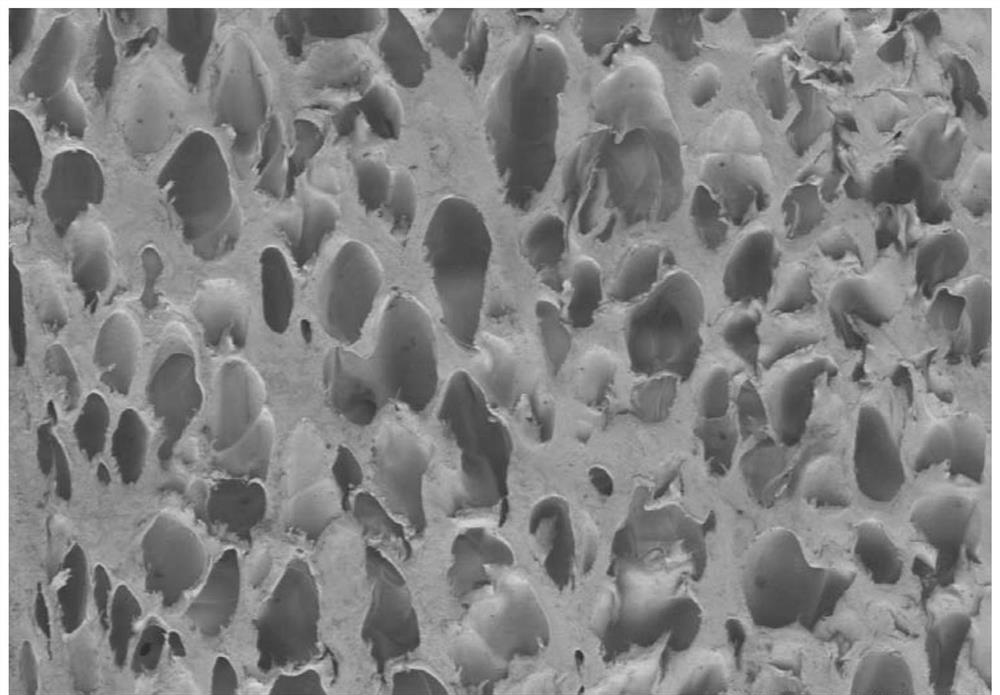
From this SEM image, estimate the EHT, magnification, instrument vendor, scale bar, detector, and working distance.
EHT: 1 kV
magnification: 0.03 K X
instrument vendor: Hitachi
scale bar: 1000000 nm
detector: SE(M)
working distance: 9.9 mm